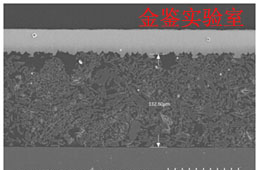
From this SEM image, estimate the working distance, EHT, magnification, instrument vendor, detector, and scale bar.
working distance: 14.7 mm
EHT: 15 kV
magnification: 0.35 K X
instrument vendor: Hitachi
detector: SE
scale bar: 100000 nm